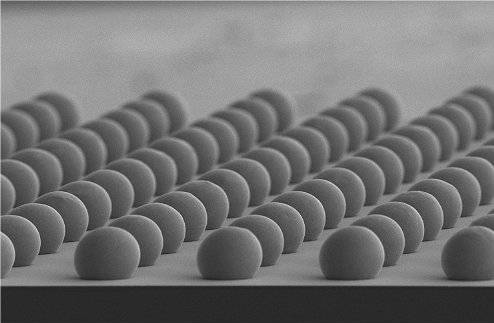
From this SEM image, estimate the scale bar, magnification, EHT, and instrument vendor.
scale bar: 100000 nm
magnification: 0.1 K X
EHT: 20 kV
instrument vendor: JEOL